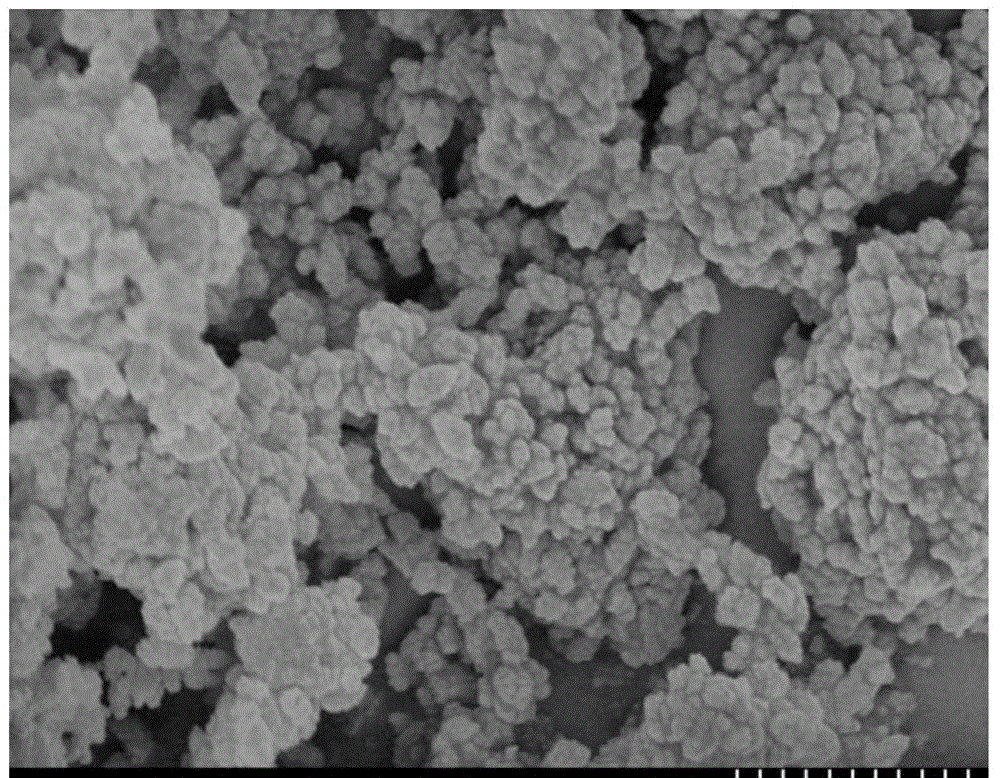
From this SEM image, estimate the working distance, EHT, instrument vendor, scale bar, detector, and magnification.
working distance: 8 mm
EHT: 5 kV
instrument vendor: Hitachi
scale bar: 1000 nm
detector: SE(U)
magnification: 30 K X